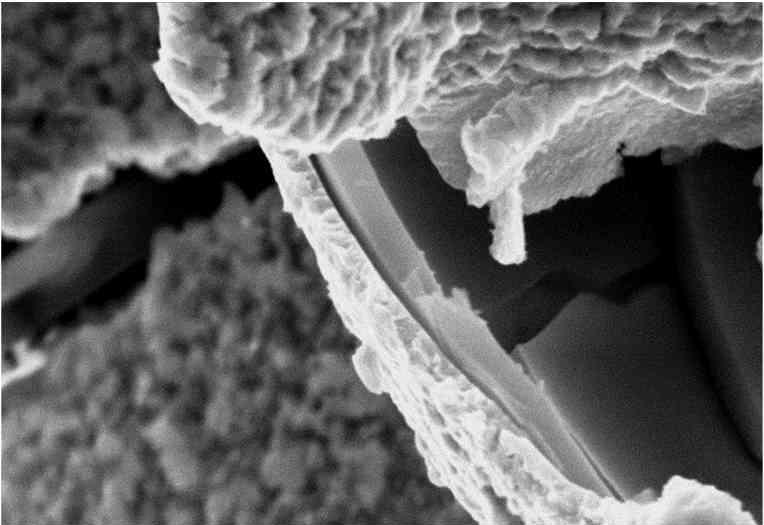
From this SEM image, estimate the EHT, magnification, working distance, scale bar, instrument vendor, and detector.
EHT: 15 kV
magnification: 10 K X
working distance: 15 mm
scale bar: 2000 nm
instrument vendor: other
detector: SE